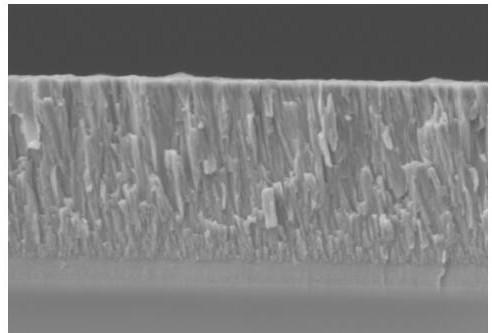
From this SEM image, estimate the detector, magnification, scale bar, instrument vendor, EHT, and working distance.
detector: SE(UL)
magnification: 30 K X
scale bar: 1000 nm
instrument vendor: Hitachi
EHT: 5 kV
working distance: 15.8 mm